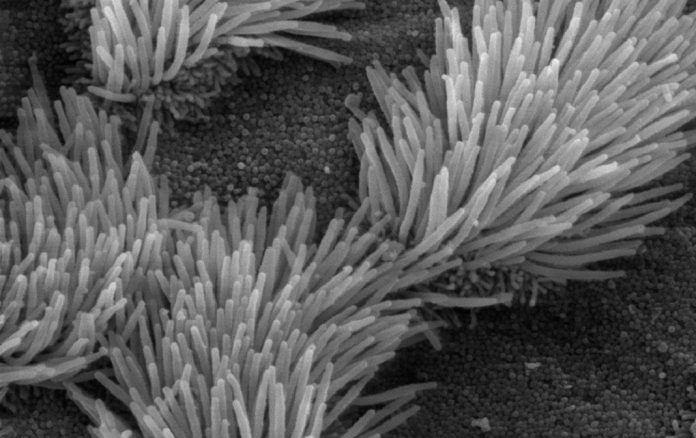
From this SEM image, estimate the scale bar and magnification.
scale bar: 5000 nm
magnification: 5 K X